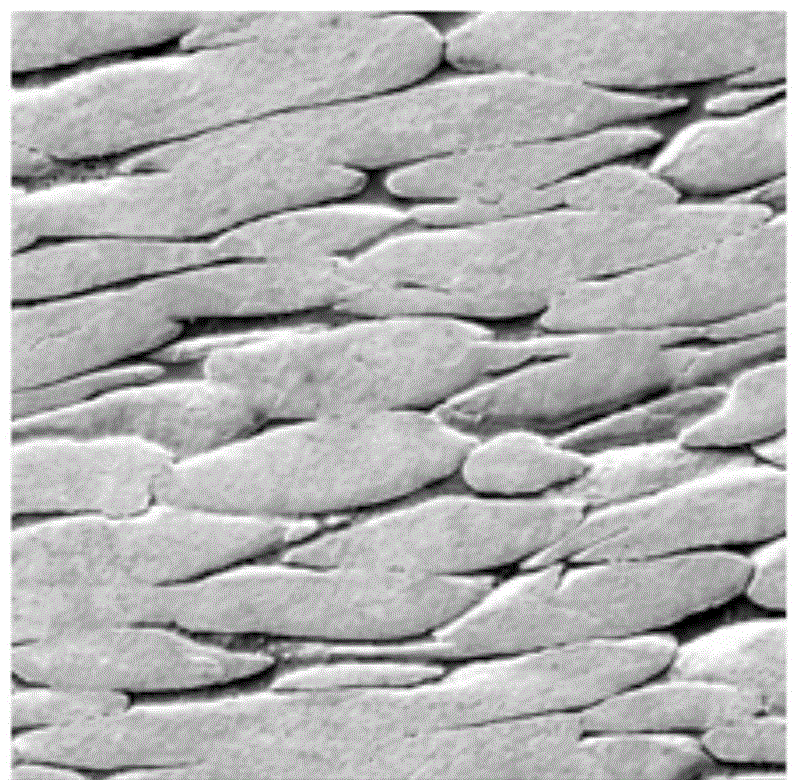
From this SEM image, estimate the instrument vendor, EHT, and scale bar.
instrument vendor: other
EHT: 20 kV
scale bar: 60000 nm